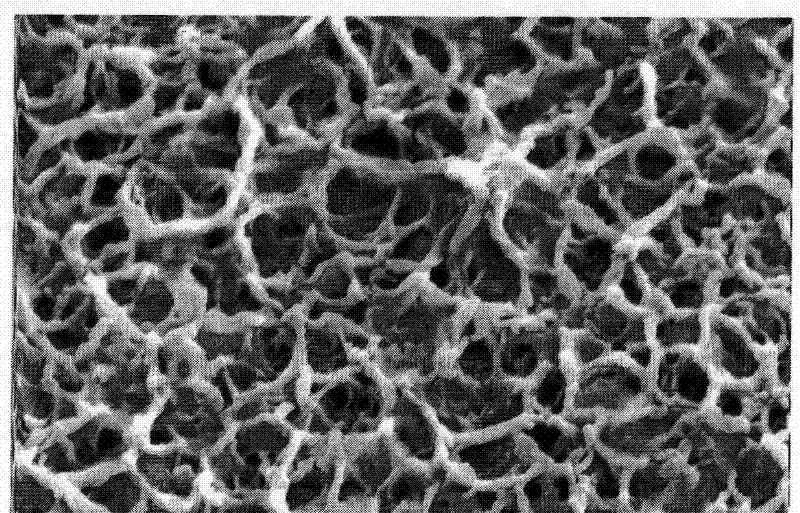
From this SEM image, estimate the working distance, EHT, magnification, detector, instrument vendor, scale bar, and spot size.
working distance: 6.4 mm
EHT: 20 kV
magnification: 30 K X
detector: SE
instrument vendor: FEI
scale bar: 2000 nm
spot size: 3